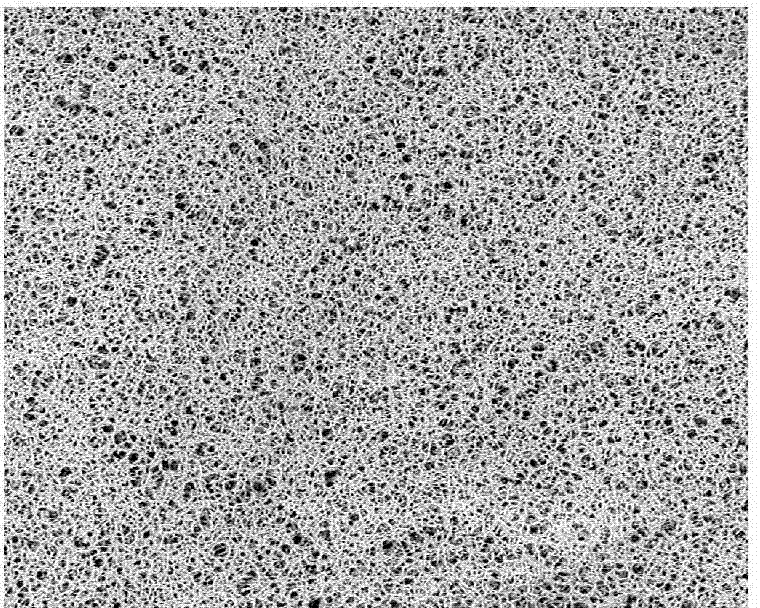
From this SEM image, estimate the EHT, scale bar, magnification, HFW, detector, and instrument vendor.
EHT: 5 kV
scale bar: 50000 nm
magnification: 1.5 K X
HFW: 179 µm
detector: BSD Full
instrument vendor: other